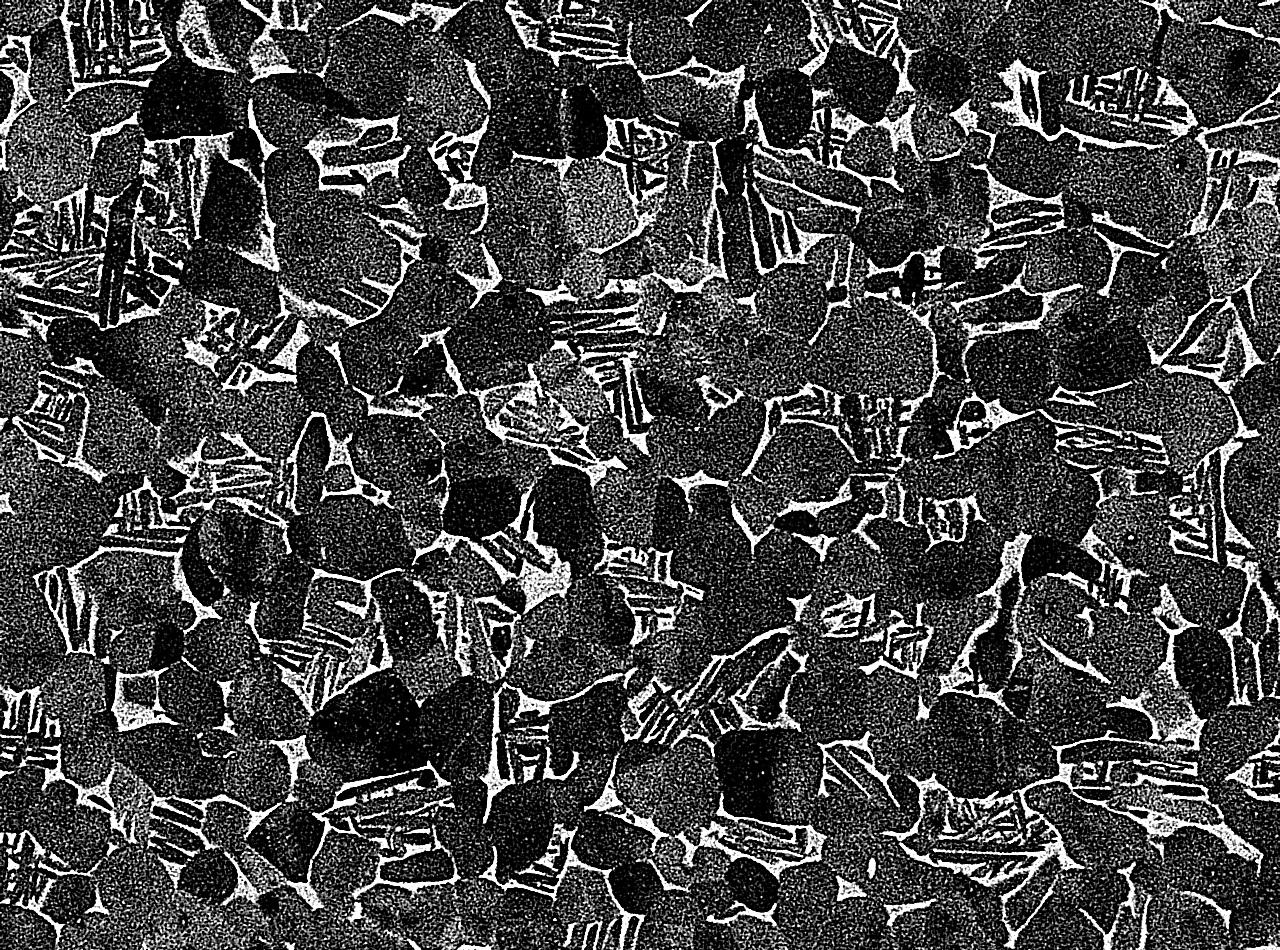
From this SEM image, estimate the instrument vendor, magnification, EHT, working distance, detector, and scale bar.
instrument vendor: JEOL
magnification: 1.1 K X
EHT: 15 kV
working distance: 10 mm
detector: COMPO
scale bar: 10000 nm